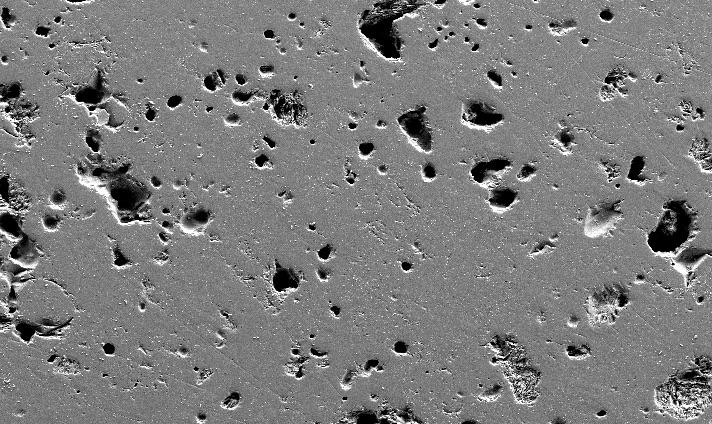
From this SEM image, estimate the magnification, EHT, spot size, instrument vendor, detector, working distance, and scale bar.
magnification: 0.2 K X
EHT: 15 kV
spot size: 3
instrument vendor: FEI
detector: SE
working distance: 4.7 mm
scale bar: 100000 nm